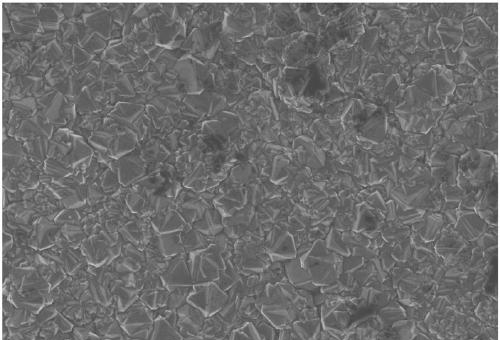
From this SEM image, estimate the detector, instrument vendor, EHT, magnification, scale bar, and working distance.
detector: InLens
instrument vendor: Zeiss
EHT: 5 kV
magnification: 3 K X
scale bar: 2000 nm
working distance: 5.5 mm